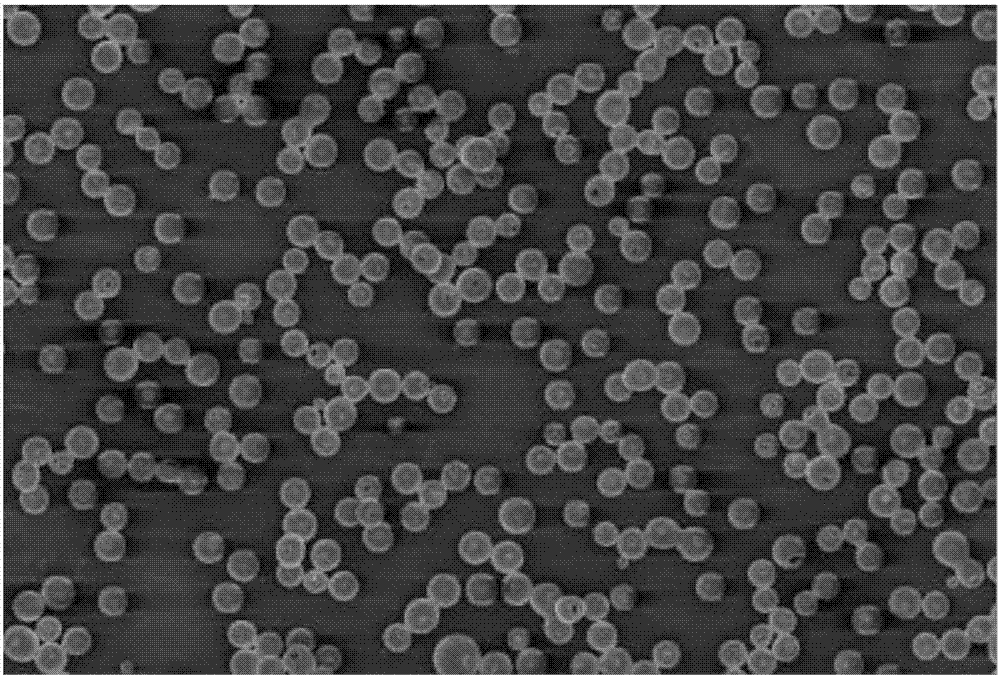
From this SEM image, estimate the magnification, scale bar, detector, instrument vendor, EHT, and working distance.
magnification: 1 K X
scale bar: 10000 nm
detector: InLens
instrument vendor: Zeiss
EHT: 5 kV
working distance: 8 mm